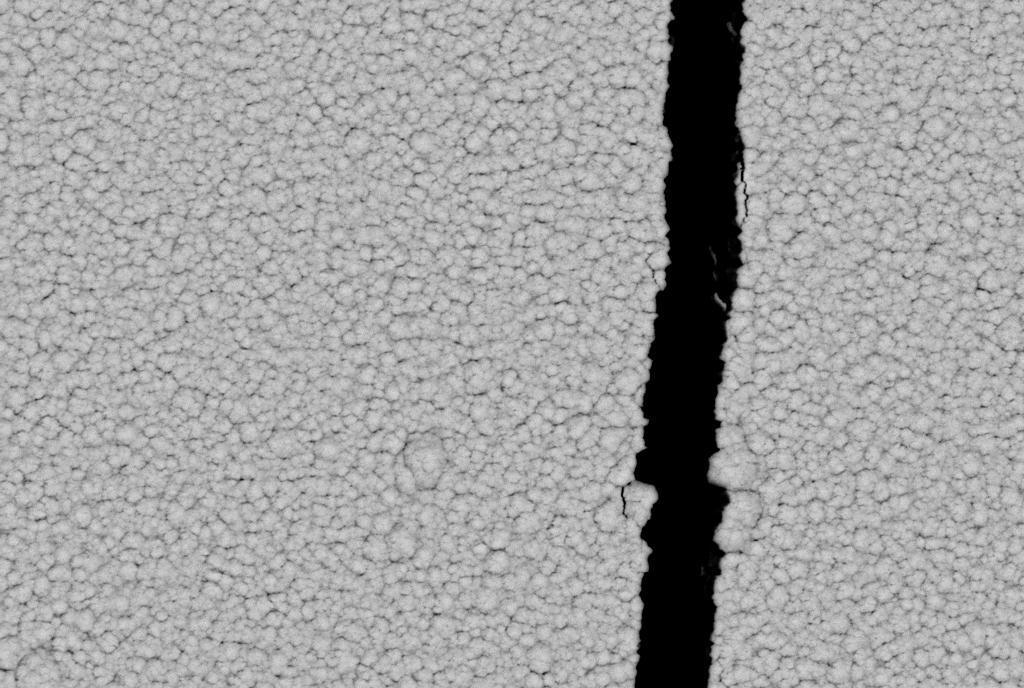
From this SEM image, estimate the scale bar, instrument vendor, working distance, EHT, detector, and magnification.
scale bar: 1000 nm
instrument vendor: Zeiss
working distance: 9 mm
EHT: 20 kV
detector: CZ BSD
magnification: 10 K X